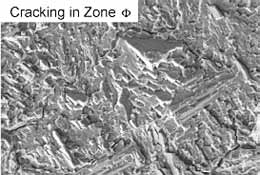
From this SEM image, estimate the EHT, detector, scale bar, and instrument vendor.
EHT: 25 kV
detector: SE1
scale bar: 10000 nm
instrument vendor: Zeiss